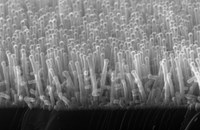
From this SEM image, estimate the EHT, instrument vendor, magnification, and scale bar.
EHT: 25 kV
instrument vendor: JEOL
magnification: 30 K X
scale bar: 1000 nm